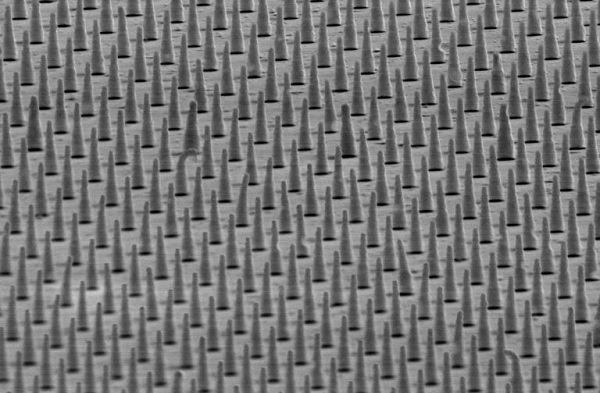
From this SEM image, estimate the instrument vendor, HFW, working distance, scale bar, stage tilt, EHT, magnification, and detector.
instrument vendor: FEI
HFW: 10.4 µm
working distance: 10.1 mm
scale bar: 3000 nm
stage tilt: -5°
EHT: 30 kV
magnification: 20 K X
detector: ETD